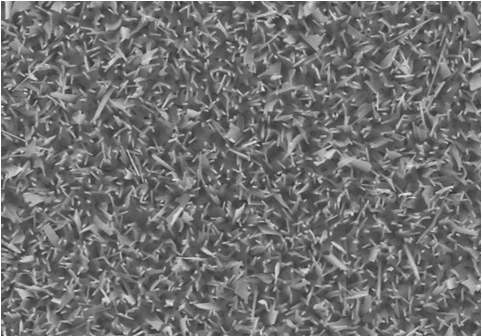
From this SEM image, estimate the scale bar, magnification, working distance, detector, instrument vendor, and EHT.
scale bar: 5000 nm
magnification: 10 K X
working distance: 12.1 mm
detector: SE(M)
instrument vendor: Hitachi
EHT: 15 kV